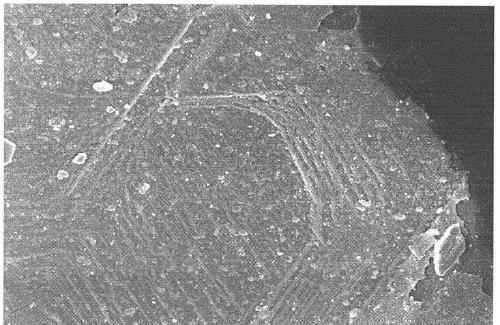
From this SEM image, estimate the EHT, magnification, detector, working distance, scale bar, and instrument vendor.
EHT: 5 kV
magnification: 5 K X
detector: SEI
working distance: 8.1 mm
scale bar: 1000 nm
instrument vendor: JEOL